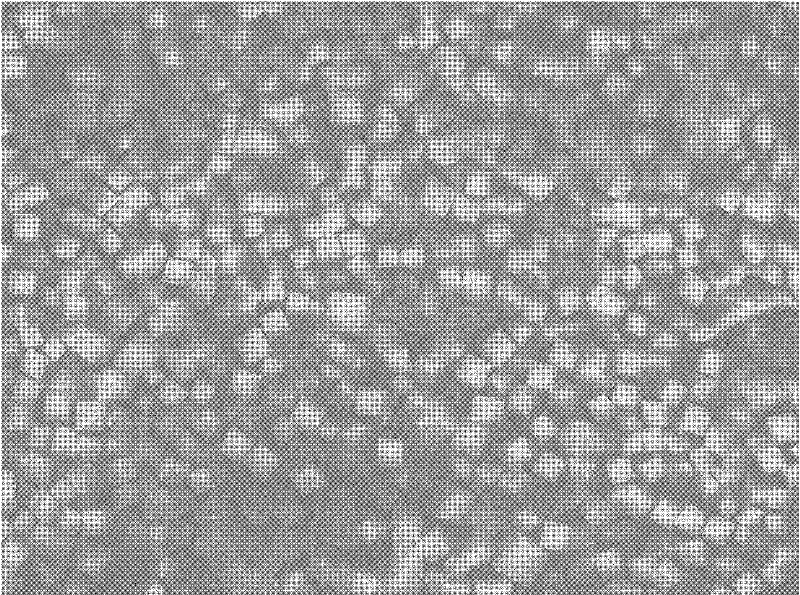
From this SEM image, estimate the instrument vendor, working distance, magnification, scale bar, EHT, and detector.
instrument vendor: JEOL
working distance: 5.9 mm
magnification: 0.35 K X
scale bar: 10000 nm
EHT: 5 kV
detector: SEI